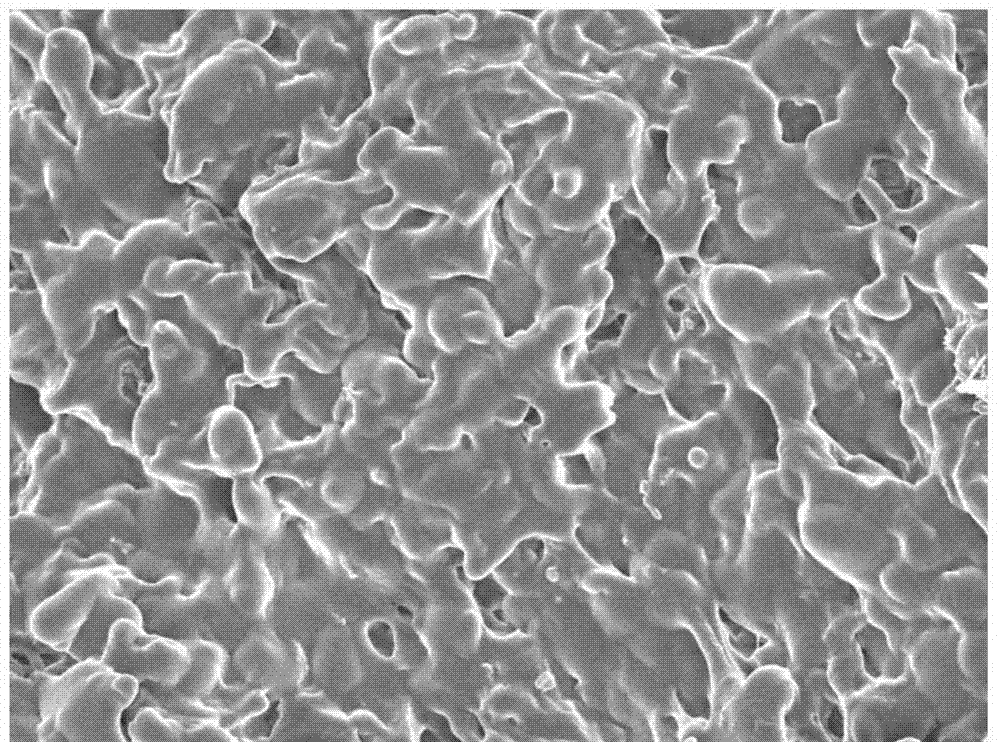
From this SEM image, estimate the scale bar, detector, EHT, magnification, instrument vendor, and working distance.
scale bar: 1000 nm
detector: SEI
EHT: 3 kV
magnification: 5 K X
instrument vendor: JEOL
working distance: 8.8 mm